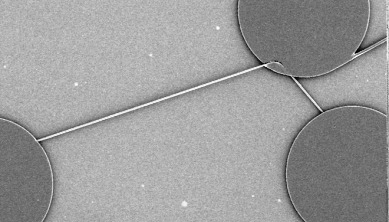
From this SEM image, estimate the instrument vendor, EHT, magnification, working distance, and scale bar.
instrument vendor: JEOL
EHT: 5 kV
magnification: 0.1 K X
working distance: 25 mm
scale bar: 100000 nm